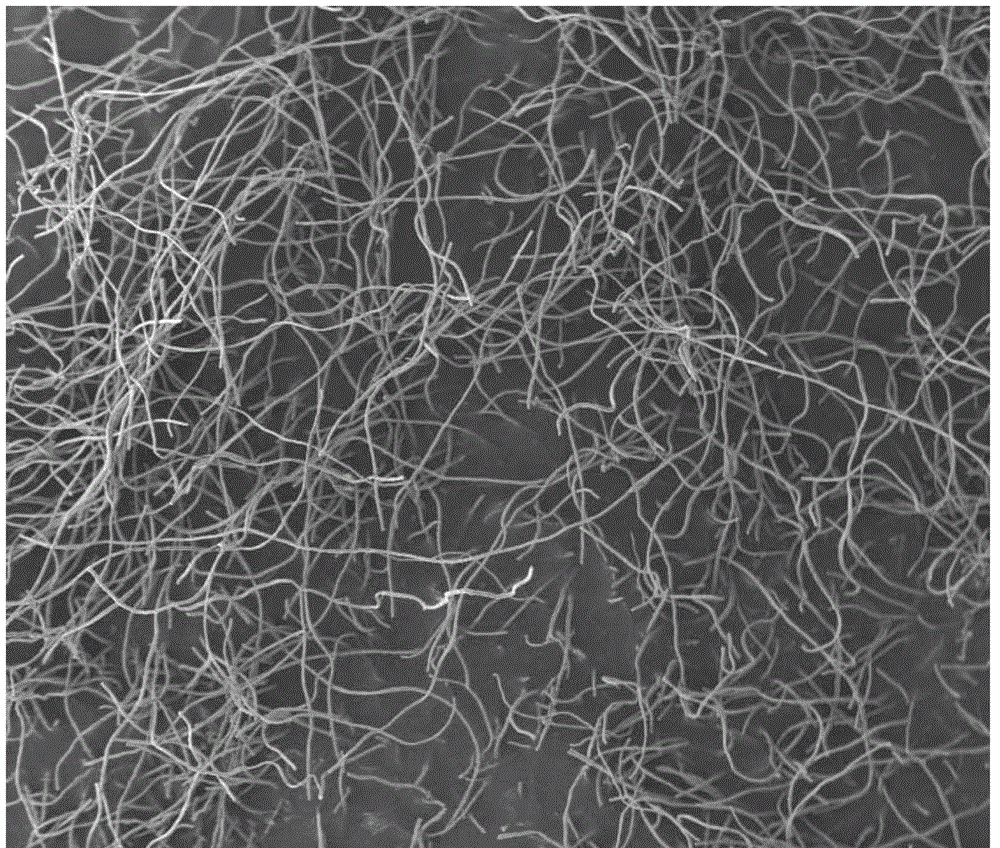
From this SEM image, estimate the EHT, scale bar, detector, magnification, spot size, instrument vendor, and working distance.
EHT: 15 kV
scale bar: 1000000 nm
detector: ETD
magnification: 0.05 K X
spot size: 6.5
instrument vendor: FEI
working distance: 9.3 mm